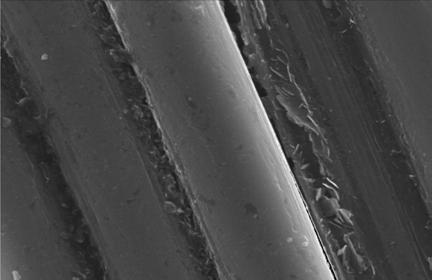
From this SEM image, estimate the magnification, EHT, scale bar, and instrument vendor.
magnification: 5 K X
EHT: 20 kV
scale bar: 2000 nm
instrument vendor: JEOL